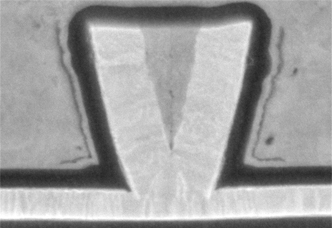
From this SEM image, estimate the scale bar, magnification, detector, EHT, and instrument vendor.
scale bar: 100 nm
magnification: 300 K X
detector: LA-BSE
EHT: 3 kV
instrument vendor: Hitachi